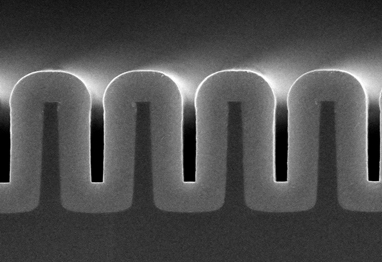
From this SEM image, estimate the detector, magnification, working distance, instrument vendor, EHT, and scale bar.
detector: SE(U,LA0)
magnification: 45 K X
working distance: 3.8 mm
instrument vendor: Hitachi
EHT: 5 kV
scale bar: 1000 nm